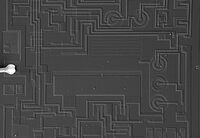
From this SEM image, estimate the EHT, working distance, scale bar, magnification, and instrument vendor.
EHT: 30 kV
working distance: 8.7 mm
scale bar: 1000000 nm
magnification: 0.06 K X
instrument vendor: JEOL